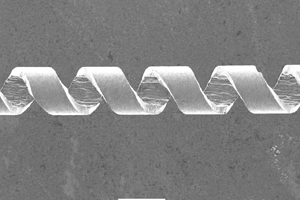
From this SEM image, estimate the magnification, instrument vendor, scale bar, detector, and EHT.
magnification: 0.2 K X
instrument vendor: JEOL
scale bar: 100000 nm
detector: SEI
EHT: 5 kV